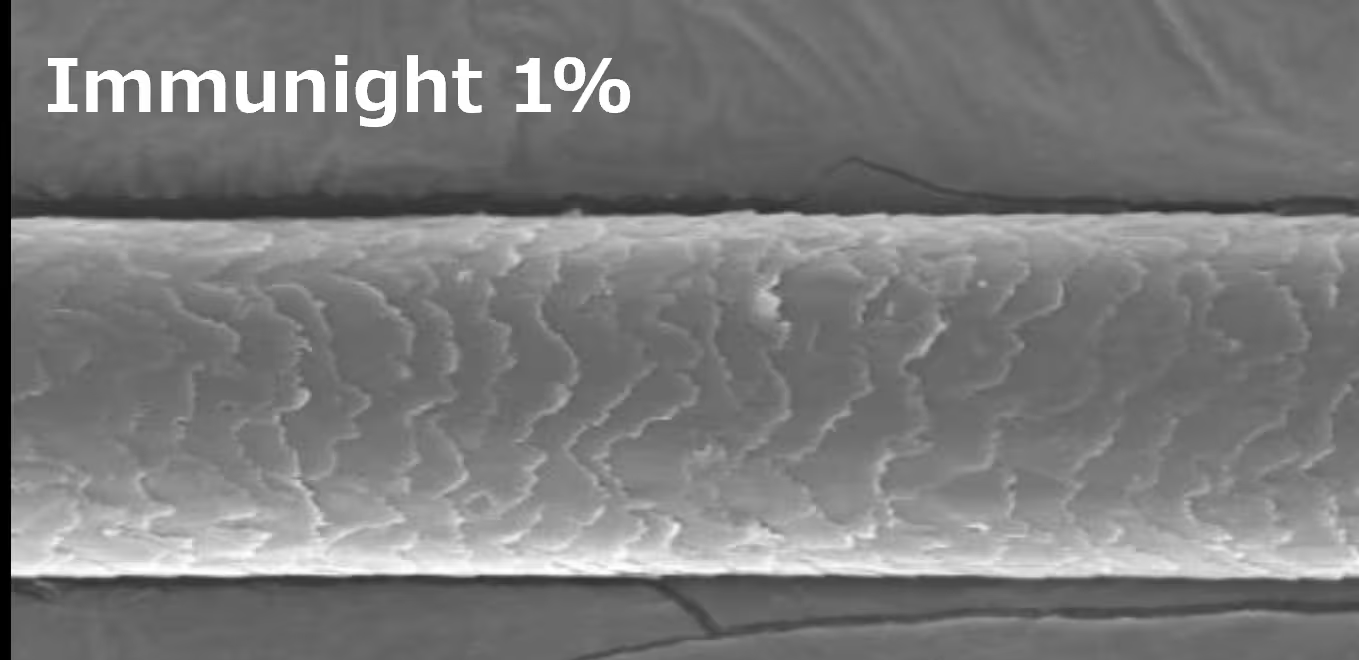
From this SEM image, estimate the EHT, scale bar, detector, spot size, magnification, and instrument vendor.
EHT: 15 kV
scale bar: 50000 nm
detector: SE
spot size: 8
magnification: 1 K X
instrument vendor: JEOL